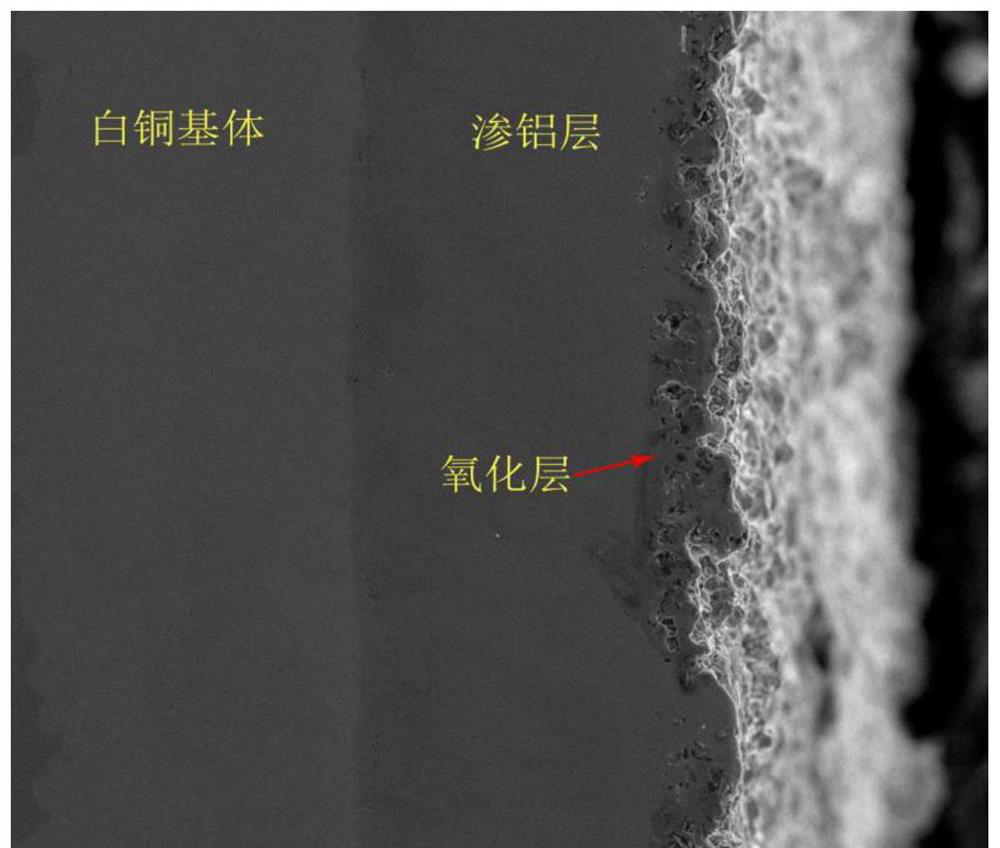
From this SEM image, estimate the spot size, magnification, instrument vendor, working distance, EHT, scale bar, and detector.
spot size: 3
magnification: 0.5 K X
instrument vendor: FEI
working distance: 8 mm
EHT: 20 kV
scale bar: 200000 nm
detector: ETD